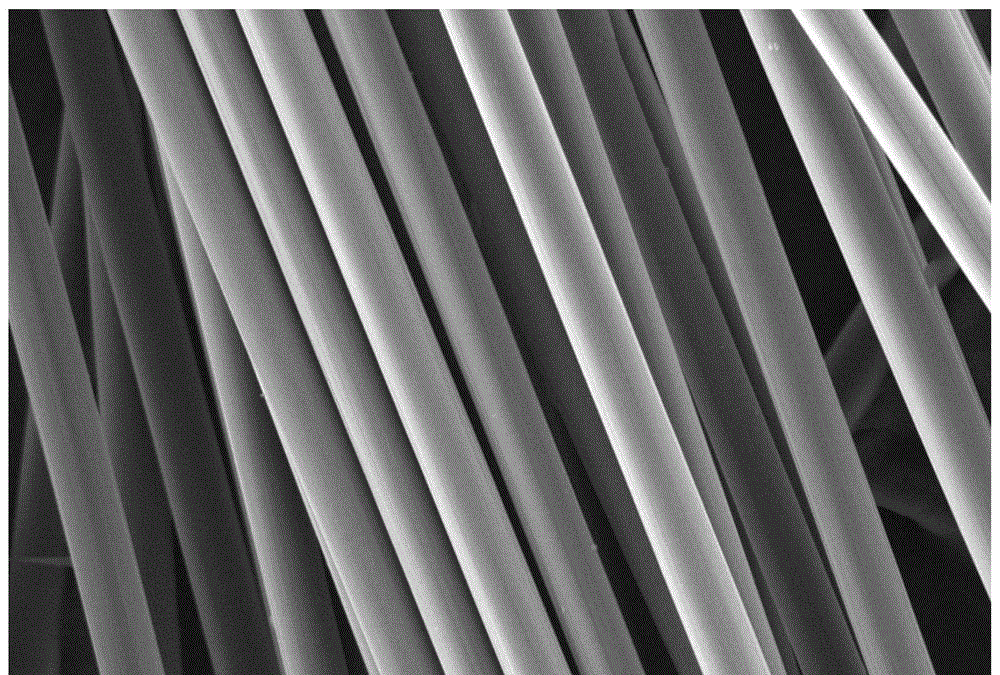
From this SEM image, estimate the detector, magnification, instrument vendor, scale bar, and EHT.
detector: SEI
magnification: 1.1 K X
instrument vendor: JEOL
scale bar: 20000 nm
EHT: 15 kV